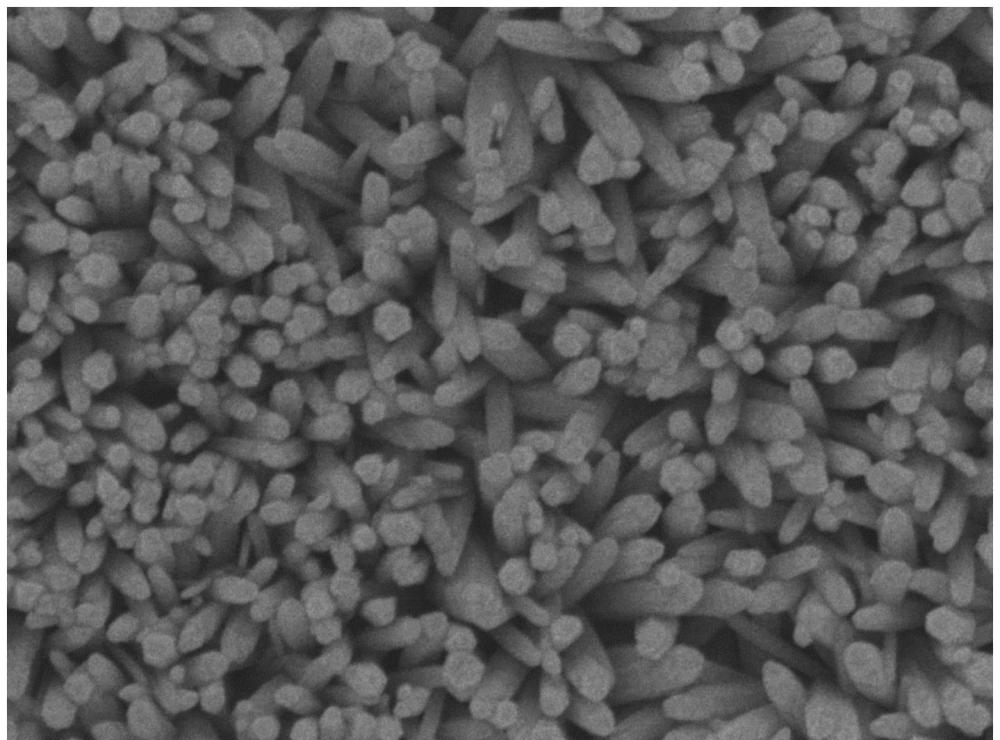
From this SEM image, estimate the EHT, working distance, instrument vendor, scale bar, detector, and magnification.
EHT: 5 kV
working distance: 7.7 mm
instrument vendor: JEOL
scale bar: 100 nm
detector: SEI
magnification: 50 K X